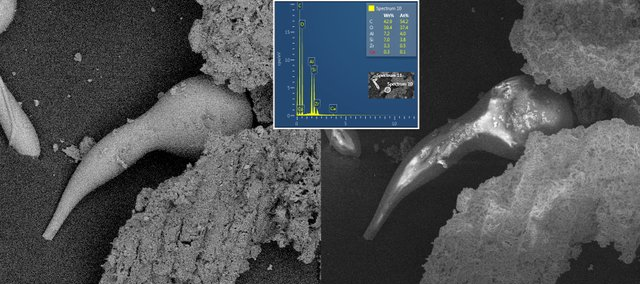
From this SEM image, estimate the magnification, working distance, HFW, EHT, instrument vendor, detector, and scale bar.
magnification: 0.5 K X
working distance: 14.97 mm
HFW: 554 µm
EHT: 15 kV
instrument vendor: Tescan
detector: BSE, SE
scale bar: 200000 nm